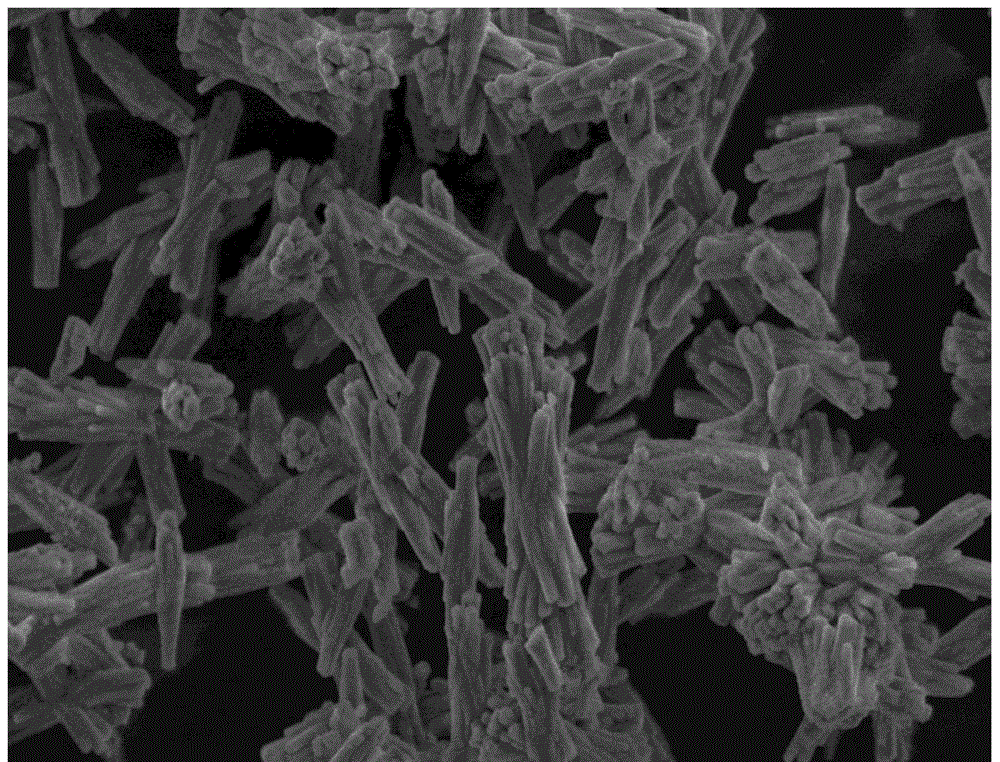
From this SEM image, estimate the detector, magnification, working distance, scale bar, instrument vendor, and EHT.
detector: ETD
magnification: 10 K X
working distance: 10.4 mm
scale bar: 10000 nm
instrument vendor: FEI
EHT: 5 kV